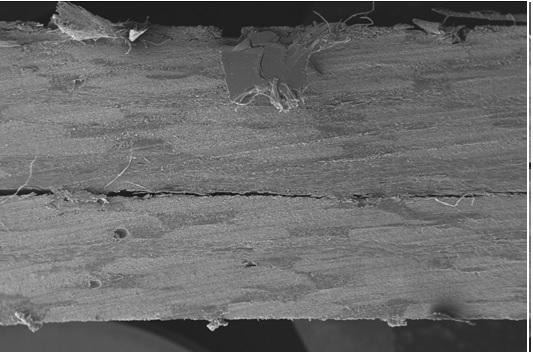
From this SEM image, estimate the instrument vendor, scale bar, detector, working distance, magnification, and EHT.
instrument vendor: Zeiss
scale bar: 200000 nm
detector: SE1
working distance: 16.5 mm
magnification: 0.015 K X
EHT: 20 kV